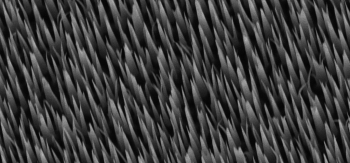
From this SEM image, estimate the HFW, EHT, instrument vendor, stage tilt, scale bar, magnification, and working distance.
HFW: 14.9 µm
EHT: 5 kV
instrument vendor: FEI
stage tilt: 20°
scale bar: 5000 nm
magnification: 20.021 K X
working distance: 10.2 mm